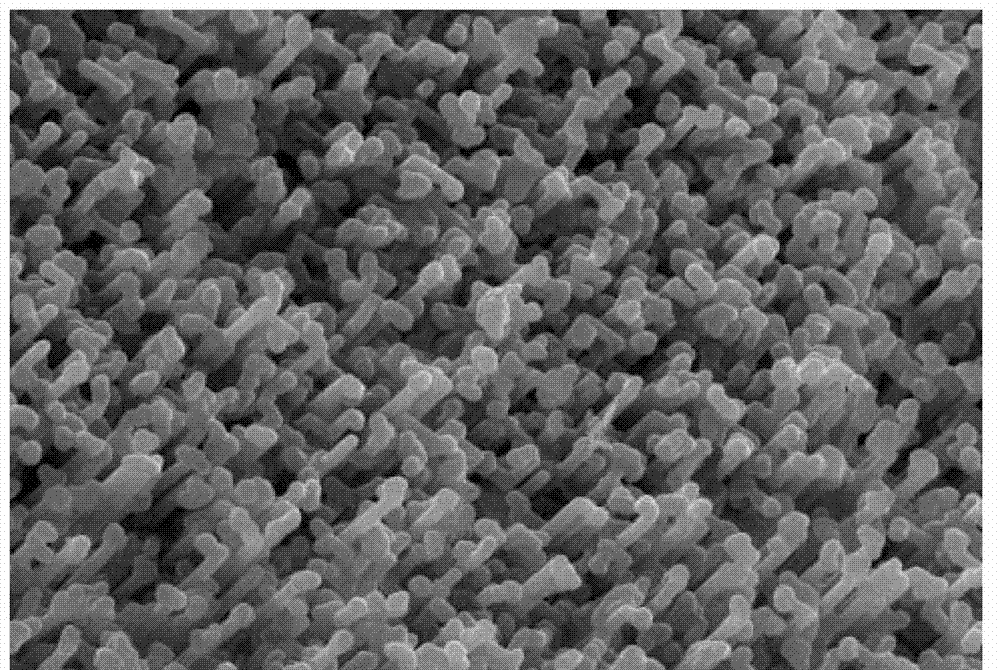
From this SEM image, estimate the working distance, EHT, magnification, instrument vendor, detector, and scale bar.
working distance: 34 mm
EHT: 30 kV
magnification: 1 K X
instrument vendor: JEOL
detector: SEI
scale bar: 10000 nm